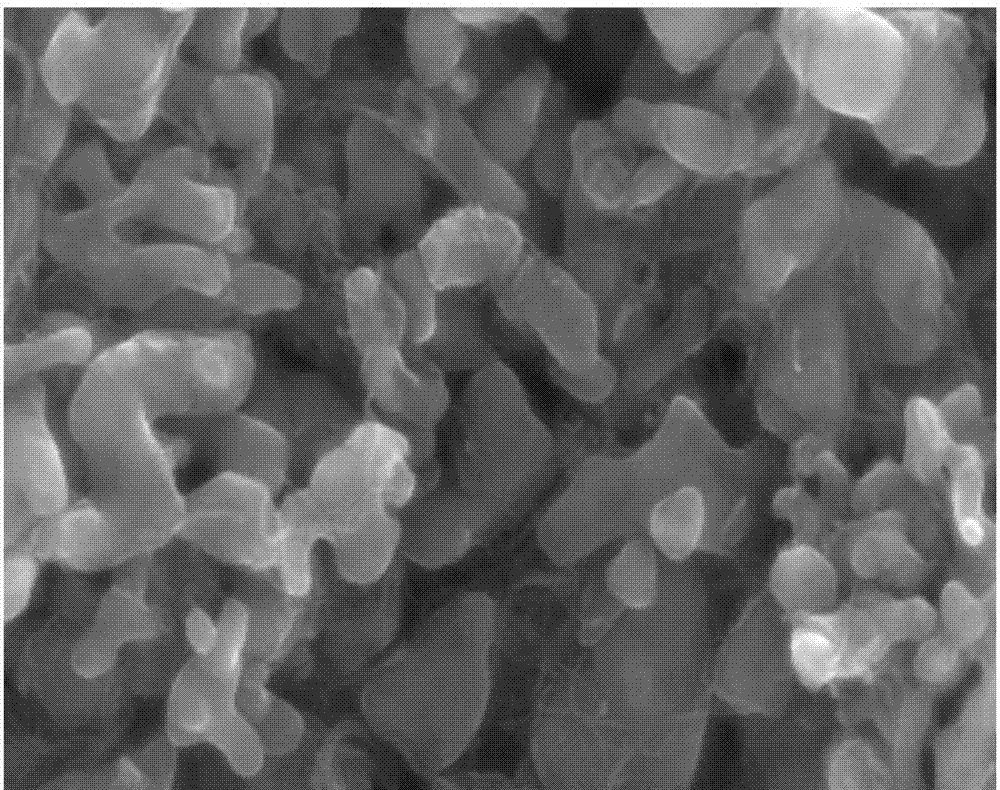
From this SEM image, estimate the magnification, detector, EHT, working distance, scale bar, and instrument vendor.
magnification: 50 K X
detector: SE(M)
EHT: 15 kV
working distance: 8 mm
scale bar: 1000 nm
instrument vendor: Hitachi